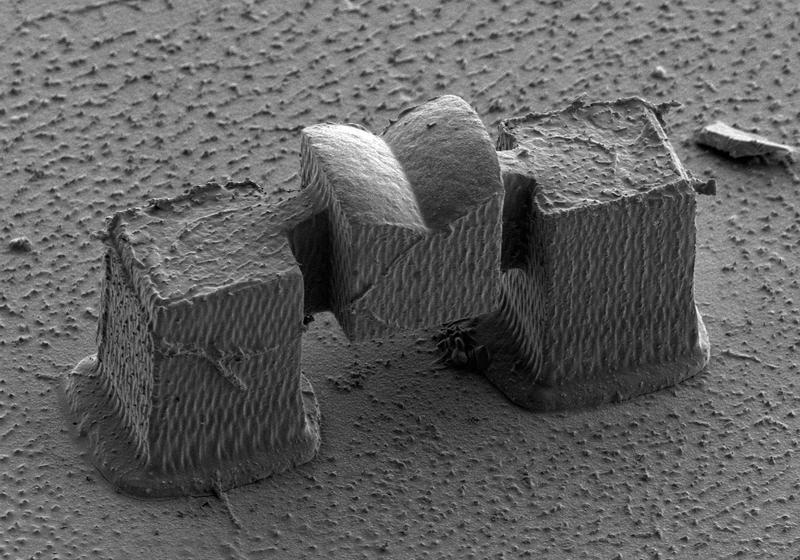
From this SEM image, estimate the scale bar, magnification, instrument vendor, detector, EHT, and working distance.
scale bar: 30000 nm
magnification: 1.5 K X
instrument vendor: Hitachi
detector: SE(L)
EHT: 1.5 kV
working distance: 16.7 mm